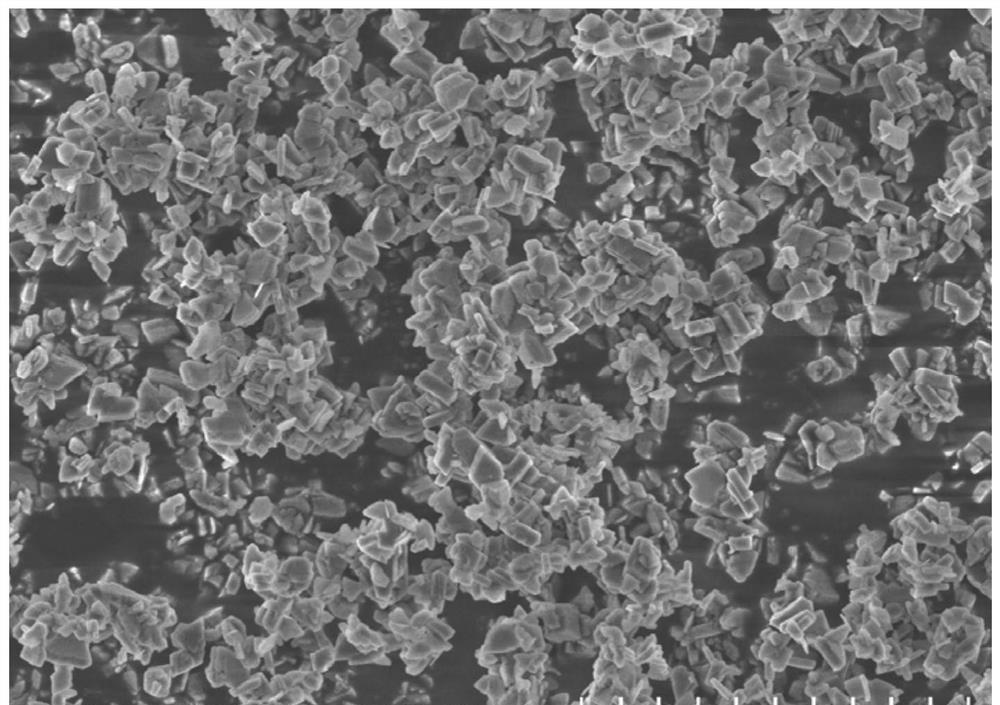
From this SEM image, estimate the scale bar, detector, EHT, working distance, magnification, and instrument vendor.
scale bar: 5000 nm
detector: SE(UL)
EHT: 3 kV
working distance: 8 mm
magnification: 10 K X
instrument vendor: Hitachi